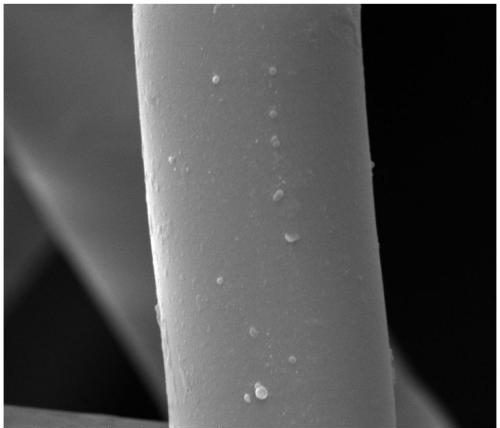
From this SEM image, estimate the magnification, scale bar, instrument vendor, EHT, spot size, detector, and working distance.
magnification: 10 K X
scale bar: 10000 nm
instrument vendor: FEI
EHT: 15 kV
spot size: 3.5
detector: ETD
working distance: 9.7 mm